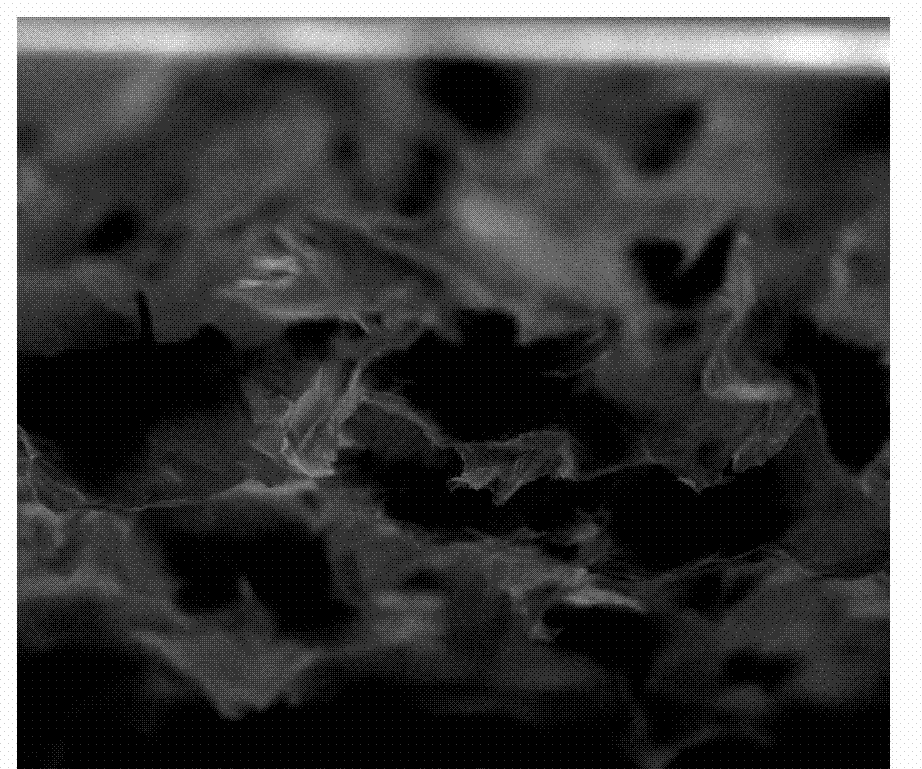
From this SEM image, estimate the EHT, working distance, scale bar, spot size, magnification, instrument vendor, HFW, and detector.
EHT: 20 kV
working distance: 11.7 mm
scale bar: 50000 nm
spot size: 3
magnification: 1.6 K X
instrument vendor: FEI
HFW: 186 µm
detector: ETD